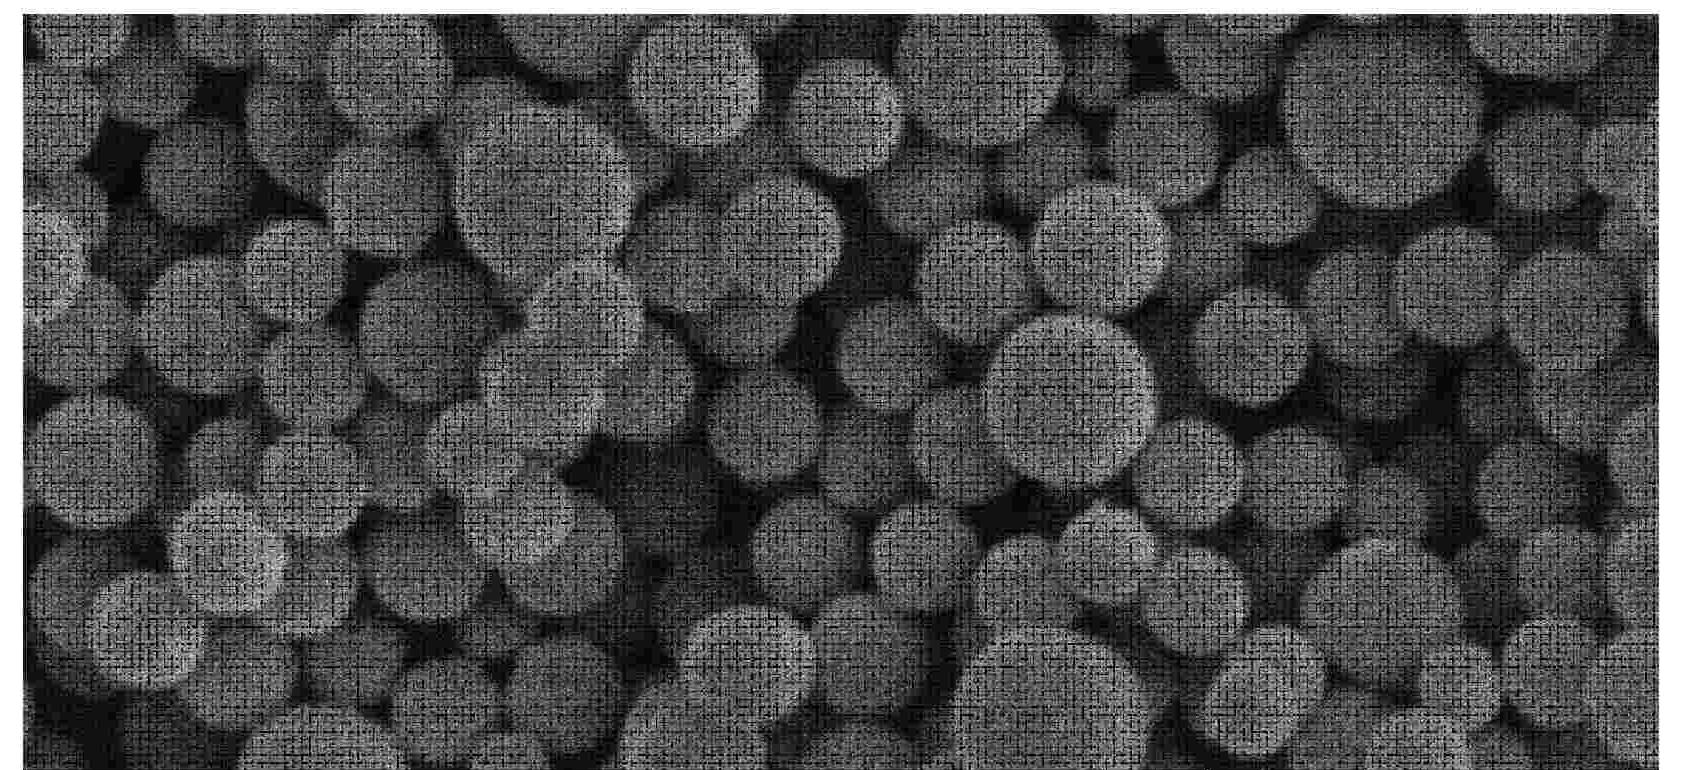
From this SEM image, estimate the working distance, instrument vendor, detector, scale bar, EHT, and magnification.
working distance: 9.5 mm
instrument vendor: Hitachi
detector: SE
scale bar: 2000 nm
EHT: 5 kV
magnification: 20 K X